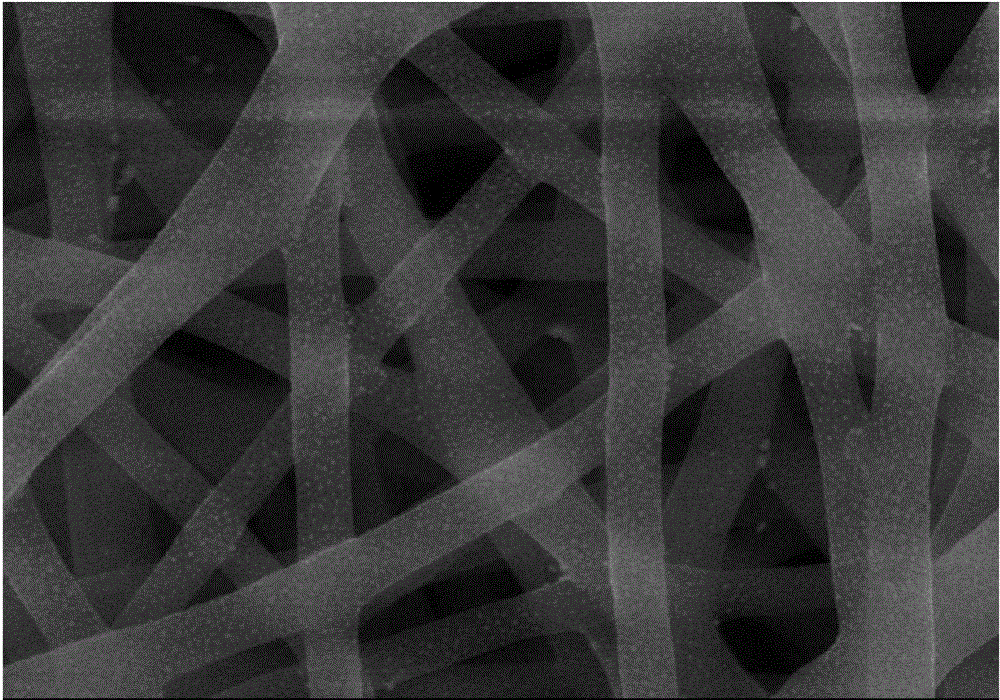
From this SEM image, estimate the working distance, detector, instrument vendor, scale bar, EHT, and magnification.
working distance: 8.3 mm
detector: SE(M)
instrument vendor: Hitachi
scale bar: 1000 nm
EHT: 10 kV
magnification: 50 K X